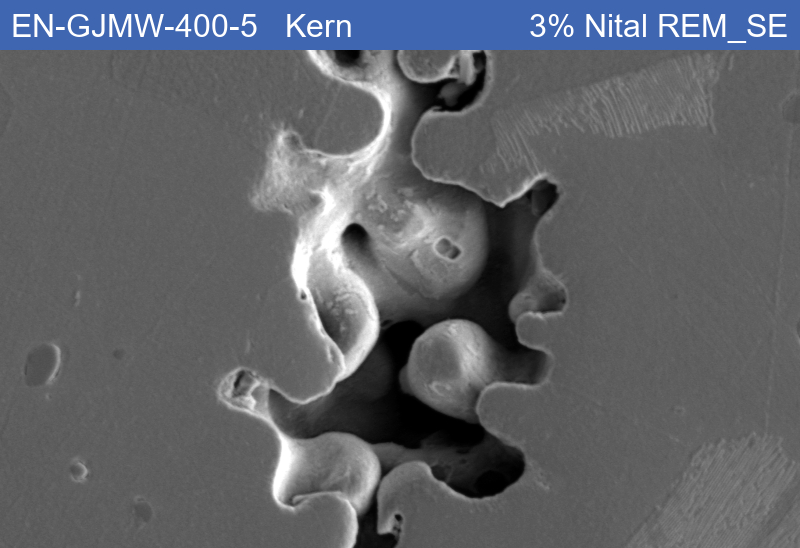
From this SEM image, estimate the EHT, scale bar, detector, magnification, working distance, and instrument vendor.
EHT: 15 kV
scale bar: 10000 nm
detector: SE1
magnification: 1 K X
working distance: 8.52 mm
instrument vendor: Zeiss